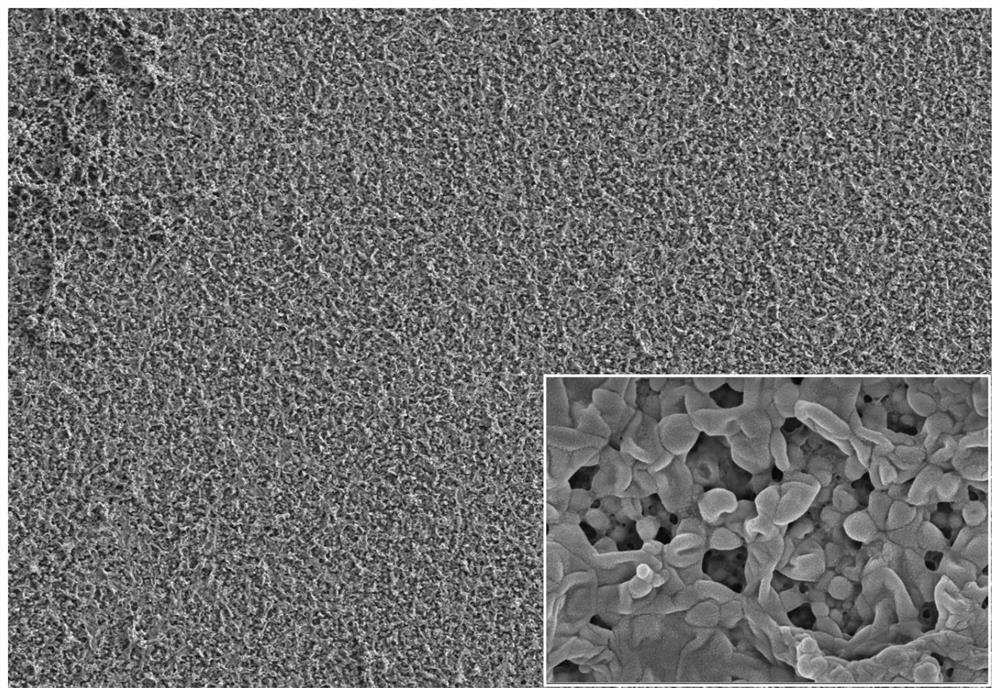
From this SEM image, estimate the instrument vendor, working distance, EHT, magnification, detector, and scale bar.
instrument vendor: Zeiss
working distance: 5.2 mm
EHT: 3 kV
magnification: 1.7 K X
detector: SE2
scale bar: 5000 nm